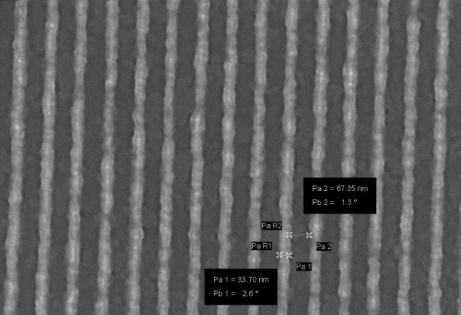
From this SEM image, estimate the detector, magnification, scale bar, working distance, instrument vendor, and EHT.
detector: InLens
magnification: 72.96 K X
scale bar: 100 nm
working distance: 7 mm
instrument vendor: Zeiss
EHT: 10 kV